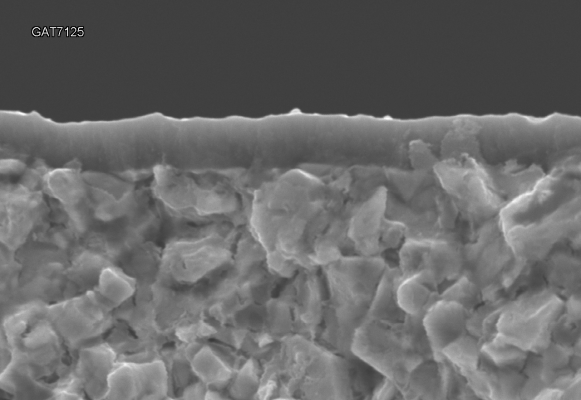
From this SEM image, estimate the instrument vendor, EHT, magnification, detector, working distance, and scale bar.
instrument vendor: Hitachi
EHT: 15 kV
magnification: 10 K X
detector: SE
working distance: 8.3 mm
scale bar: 5000 nm